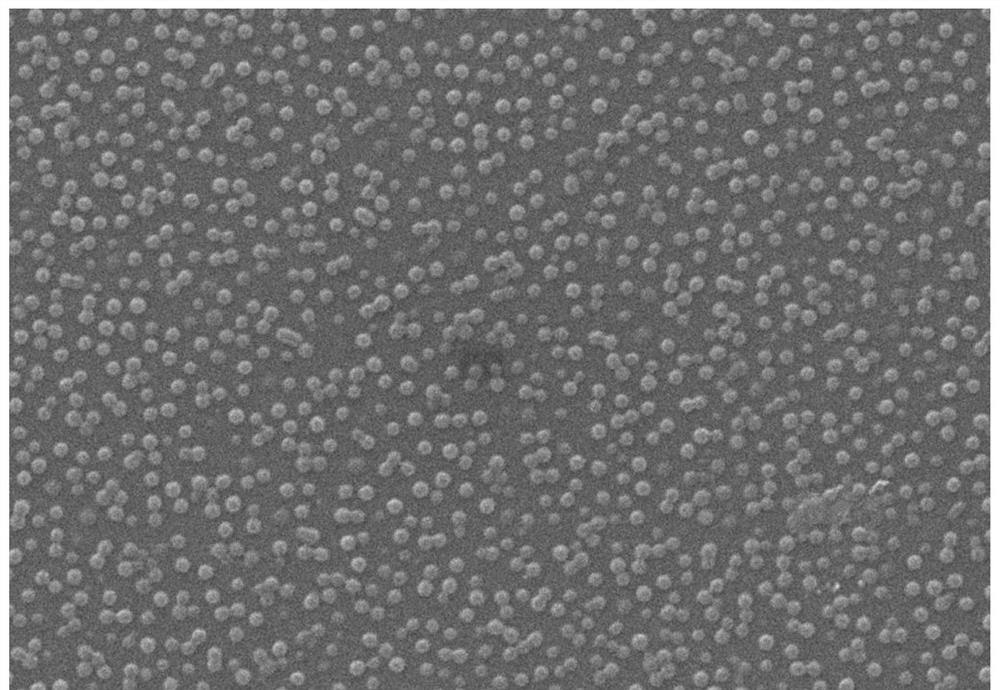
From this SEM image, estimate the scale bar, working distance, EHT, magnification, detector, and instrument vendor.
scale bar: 5000 nm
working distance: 4.9 mm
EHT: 15 kV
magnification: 10 K X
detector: SE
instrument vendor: Hitachi